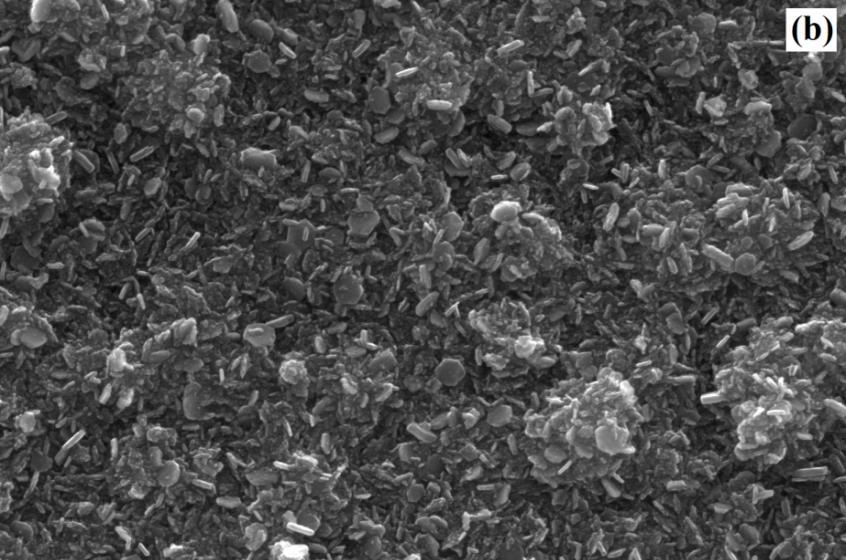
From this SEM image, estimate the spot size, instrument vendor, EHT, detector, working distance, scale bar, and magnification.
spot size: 3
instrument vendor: FEI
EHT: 20 kV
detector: ETD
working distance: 11.7 mm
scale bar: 1000 nm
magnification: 50 K X